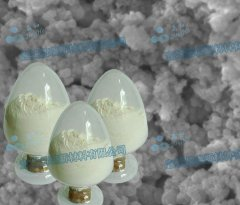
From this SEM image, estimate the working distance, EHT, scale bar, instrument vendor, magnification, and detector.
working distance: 2.9 mm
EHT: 5 kV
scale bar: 100 nm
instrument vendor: JEOL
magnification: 150 K X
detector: SEI